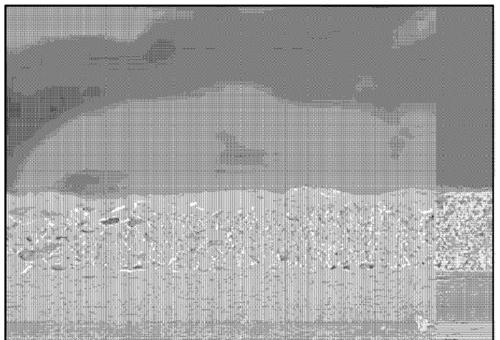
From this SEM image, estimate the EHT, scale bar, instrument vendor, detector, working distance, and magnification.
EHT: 5 kV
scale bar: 20000 nm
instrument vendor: Hitachi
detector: SE(M)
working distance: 8.7 mm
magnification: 2.5 K X